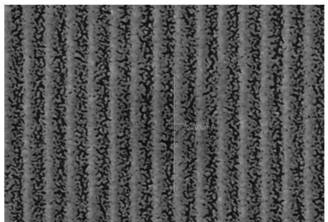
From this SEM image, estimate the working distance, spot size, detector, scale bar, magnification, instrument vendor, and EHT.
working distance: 10 mm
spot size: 50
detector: SEI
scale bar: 1000 nm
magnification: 15 K X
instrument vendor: JEOL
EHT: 15 kV